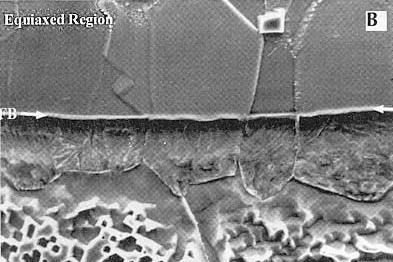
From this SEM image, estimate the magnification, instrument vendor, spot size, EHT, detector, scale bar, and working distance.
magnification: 1.6 K X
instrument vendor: FEI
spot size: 4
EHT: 20 kV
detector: SE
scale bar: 20000 nm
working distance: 10 mm